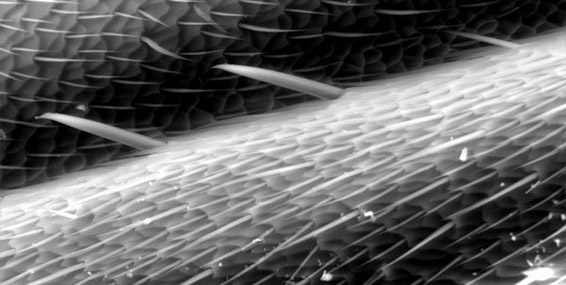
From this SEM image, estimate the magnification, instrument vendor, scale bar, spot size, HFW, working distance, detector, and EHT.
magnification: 0.8 K X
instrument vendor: FEI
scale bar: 50000 nm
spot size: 4.5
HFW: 259 µm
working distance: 7.6 mm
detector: GSED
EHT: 20 kV